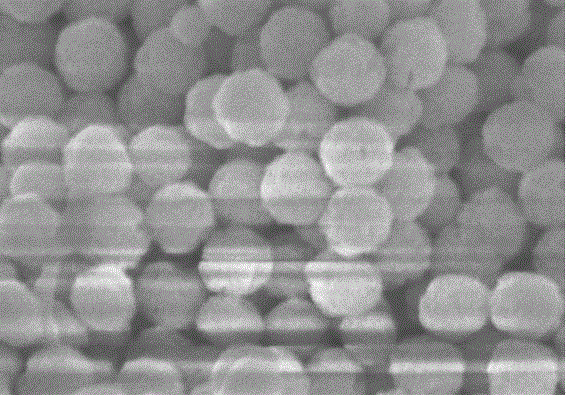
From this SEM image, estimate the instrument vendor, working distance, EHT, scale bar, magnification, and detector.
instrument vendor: Hitachi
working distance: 9.2 mm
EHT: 5 kV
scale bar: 500 nm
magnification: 100 K X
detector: SE(M)